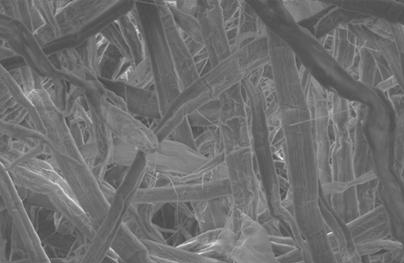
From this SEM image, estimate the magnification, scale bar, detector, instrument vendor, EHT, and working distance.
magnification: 1.32 K X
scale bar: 20000 nm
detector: SE1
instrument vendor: Zeiss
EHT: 11 kV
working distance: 17 mm